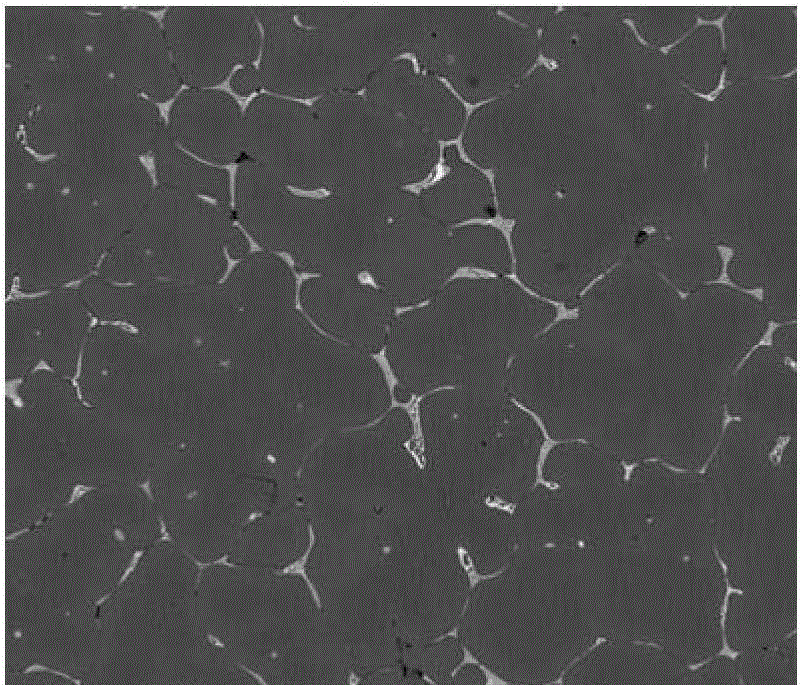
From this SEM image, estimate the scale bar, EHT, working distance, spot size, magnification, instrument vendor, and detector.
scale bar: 200000 nm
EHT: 20 kV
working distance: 10.2 mm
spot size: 6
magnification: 0.5 K X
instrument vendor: FEI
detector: DualBSD